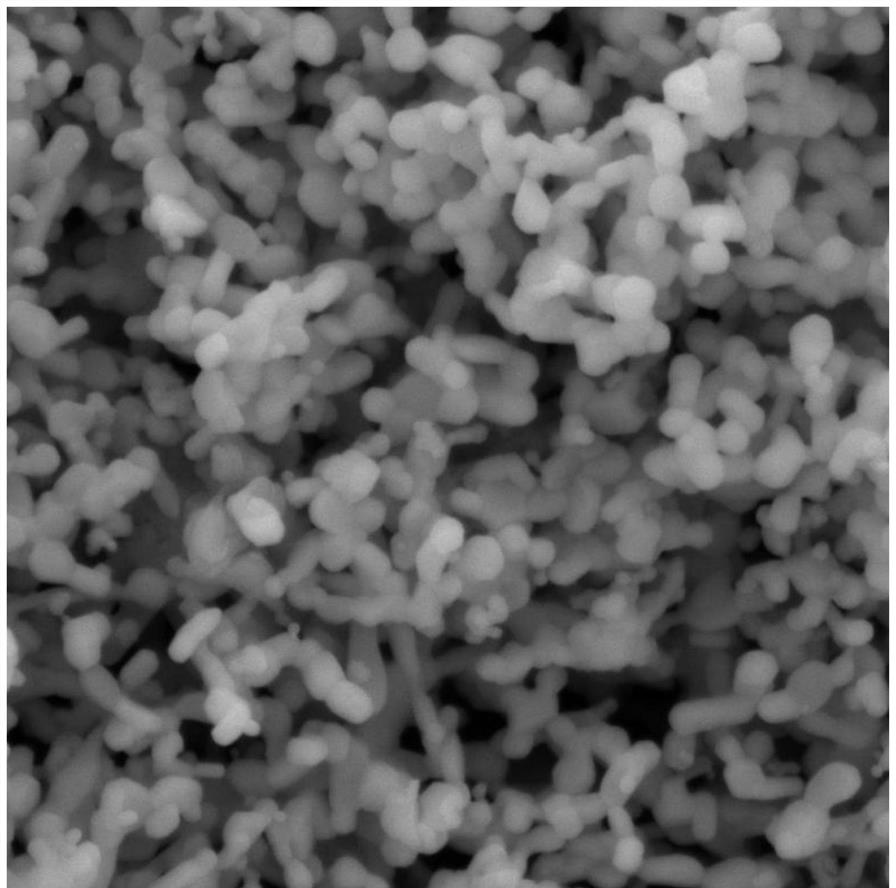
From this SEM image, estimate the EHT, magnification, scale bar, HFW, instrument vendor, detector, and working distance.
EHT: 10 kV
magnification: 50 K X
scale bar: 1000 nm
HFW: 4.15 µm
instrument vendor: Tescan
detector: SE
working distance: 9.8 mm